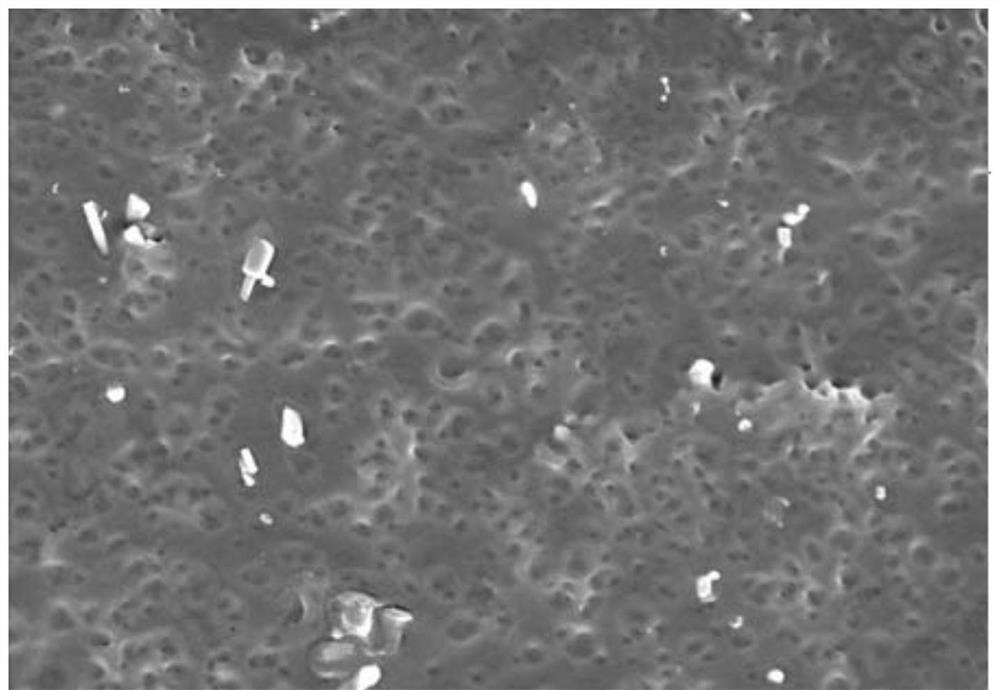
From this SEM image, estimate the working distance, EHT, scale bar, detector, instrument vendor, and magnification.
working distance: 8 mm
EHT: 5 kV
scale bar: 5000 nm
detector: SE(M)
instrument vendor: Hitachi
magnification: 10 K X